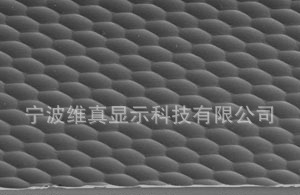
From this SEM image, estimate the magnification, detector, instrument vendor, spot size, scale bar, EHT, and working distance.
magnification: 0.2 K X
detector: SE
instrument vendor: FEI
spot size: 5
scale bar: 200000 nm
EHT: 20 kV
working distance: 61.5 mm